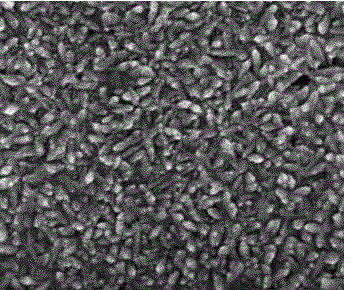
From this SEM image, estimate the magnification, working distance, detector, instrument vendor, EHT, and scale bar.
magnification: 200 K X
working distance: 5.1 mm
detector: TLD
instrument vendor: FEI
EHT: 15 kV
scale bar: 500 nm